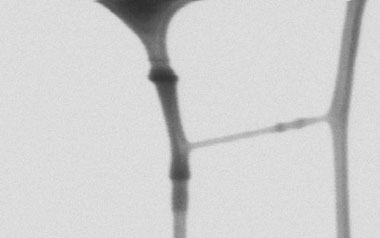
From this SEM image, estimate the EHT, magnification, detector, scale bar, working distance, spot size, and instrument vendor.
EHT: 15 kV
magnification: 120 K X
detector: SE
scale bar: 500 nm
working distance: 18.4 mm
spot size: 3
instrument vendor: FEI